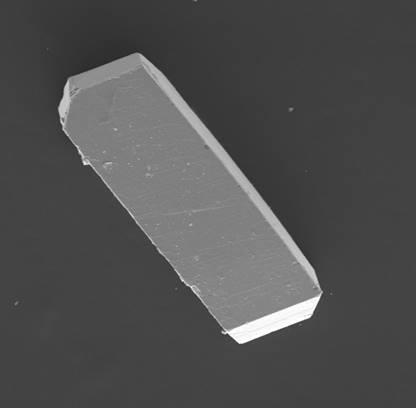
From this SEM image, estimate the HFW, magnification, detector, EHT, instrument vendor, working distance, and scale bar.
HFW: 931 µm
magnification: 0.3 K X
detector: SE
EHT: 20 kV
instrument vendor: Tescan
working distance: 11.96 mm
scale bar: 200000 nm